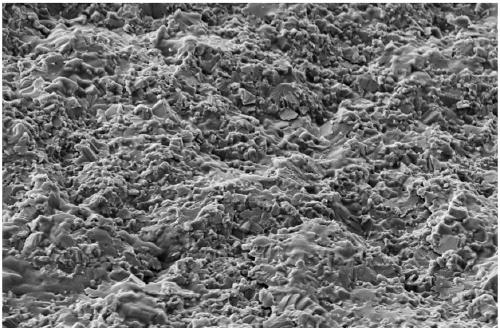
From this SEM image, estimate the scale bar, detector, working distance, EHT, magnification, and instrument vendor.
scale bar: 2000 nm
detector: SE2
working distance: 11.3 mm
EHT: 5 kV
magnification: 4 K X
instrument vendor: Zeiss